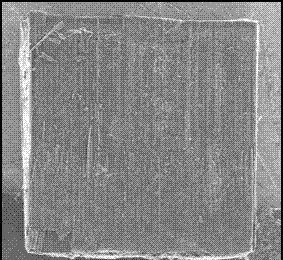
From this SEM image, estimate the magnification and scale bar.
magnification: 0.025 K X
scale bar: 1000000 nm